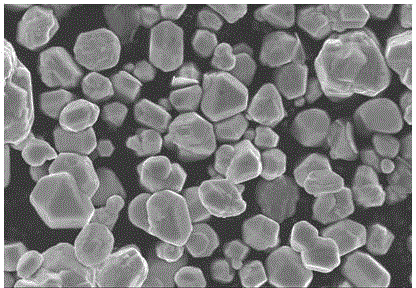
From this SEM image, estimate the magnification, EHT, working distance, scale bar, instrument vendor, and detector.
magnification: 10 K X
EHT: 10 kV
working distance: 7.6 mm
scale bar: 5000 nm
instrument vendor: Hitachi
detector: SE(UL)